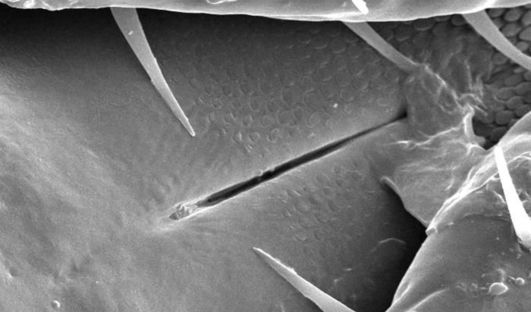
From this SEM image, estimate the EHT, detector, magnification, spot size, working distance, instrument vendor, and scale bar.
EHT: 30 kV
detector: SE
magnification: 0.801 K X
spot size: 3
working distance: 7.1 mm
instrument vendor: FEI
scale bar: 20000 nm